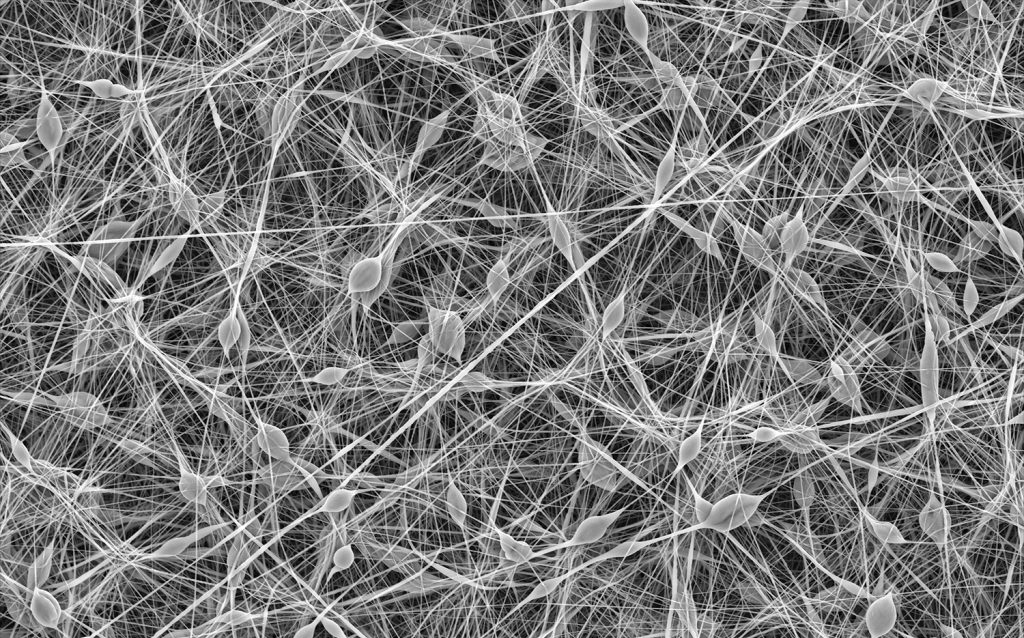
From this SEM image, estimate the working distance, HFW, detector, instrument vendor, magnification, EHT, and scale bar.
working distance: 4.649 mm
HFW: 208 µm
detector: Mix 50%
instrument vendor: Thermo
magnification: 2.5 K X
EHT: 10 kV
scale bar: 50000 nm